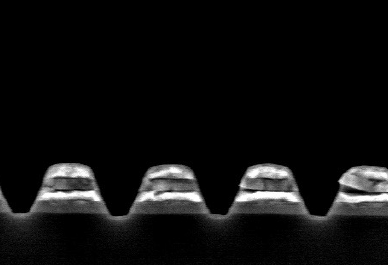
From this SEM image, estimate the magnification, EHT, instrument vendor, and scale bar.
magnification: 40 K X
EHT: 5 kV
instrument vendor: JEOL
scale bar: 100 nm